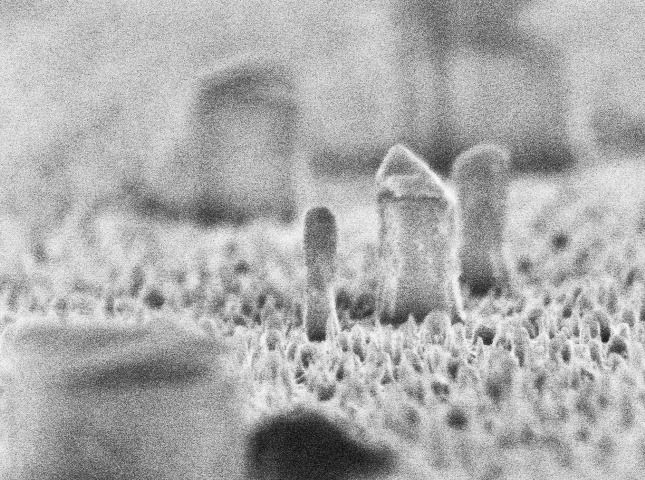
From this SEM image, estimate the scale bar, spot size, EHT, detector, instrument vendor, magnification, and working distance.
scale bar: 500 nm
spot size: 2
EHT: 10 kV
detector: TLD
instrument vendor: FEI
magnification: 48.017 K X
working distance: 5.4 mm